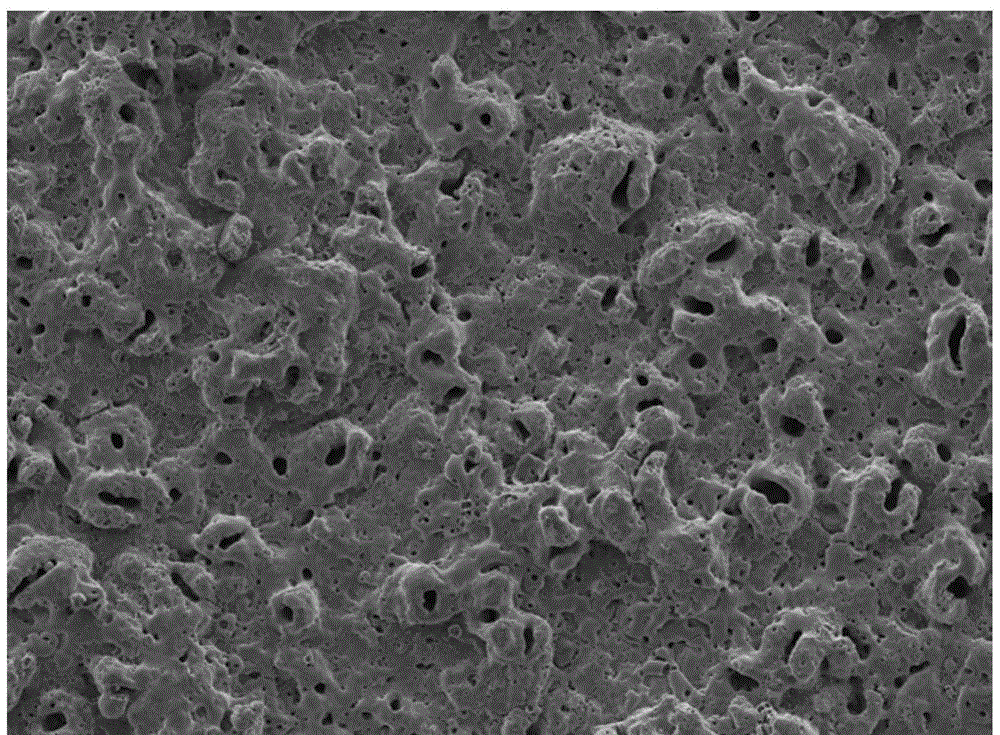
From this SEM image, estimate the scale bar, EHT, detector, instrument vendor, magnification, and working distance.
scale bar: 100000 nm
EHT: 12 kV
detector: SEI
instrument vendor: JEOL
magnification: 0.2 K X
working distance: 8 mm